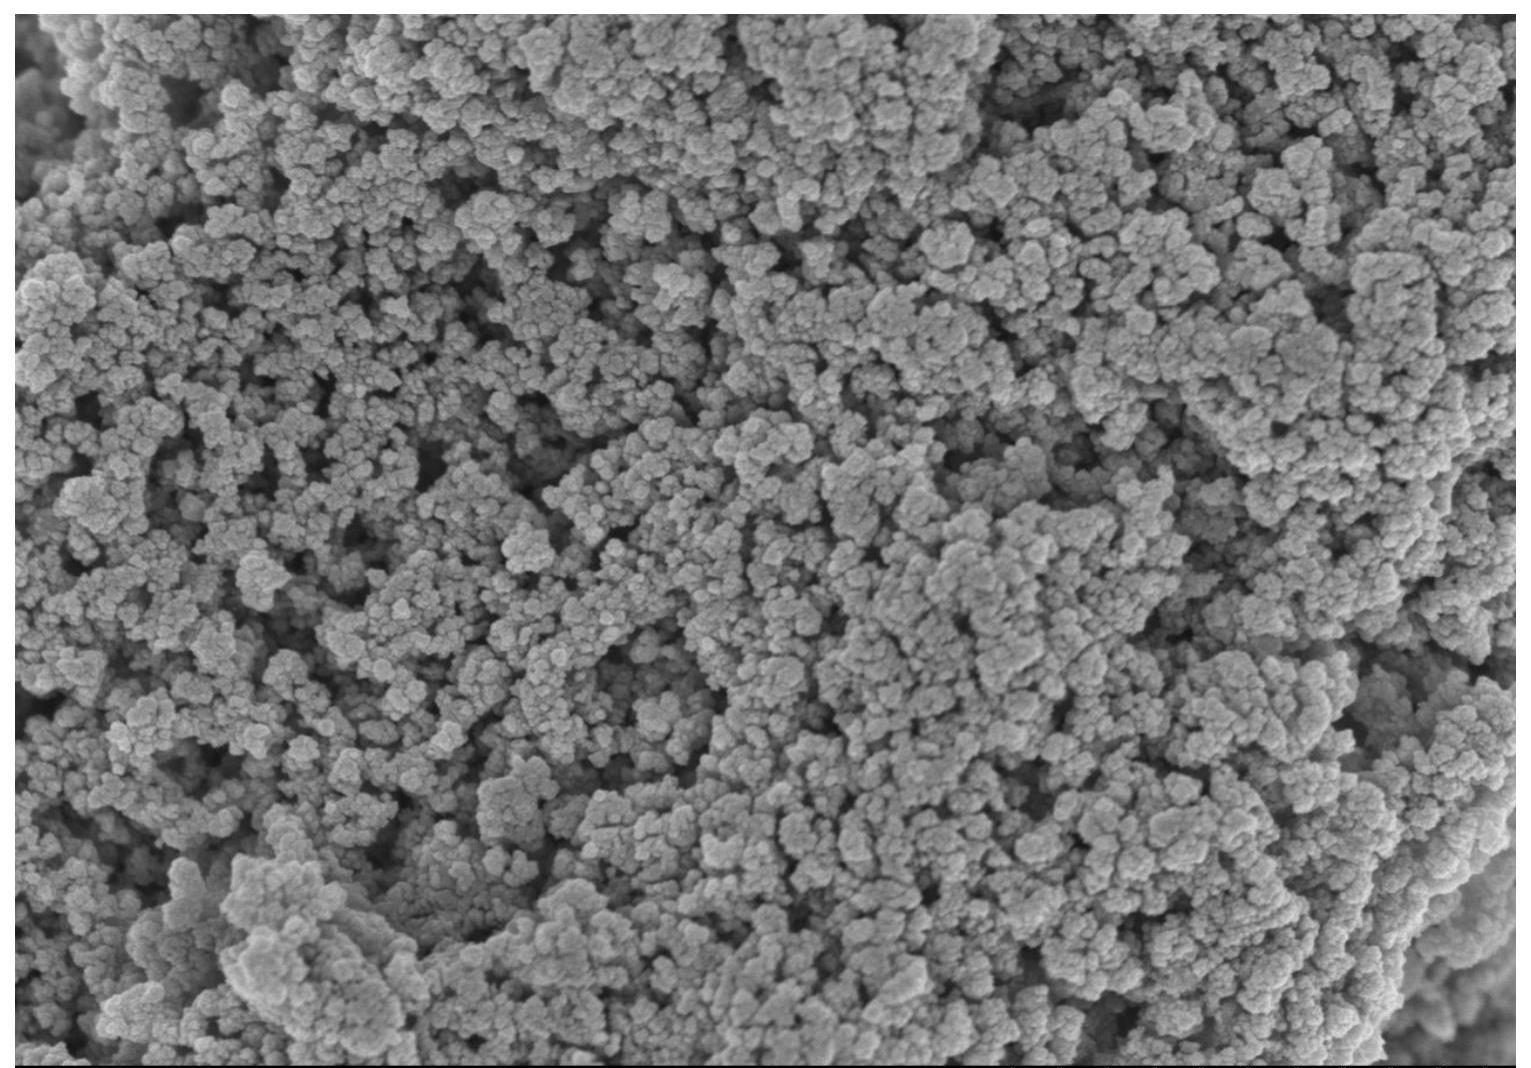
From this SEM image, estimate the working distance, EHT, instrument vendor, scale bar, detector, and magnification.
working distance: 4.2 mm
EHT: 3 kV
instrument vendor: Hitachi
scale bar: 2000 nm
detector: SE(M)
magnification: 20 K X